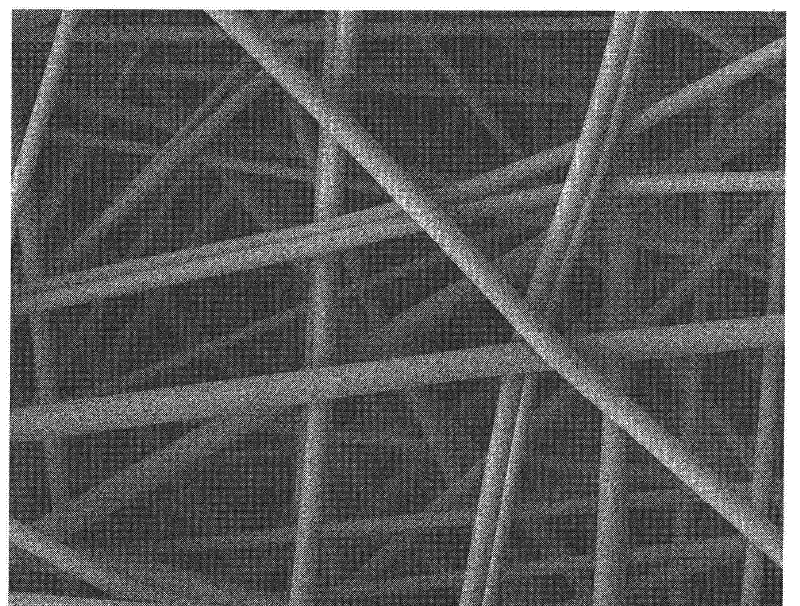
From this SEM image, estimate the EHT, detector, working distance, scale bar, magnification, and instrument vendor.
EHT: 5 kV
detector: LEI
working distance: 9.2 mm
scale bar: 1000 nm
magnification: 10 K X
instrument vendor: JEOL